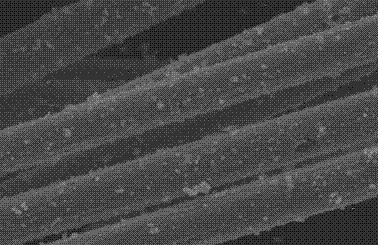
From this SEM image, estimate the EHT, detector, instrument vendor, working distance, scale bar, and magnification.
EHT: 22 kV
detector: SE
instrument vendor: FEI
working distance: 9 mm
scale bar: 10000 nm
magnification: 2 K X